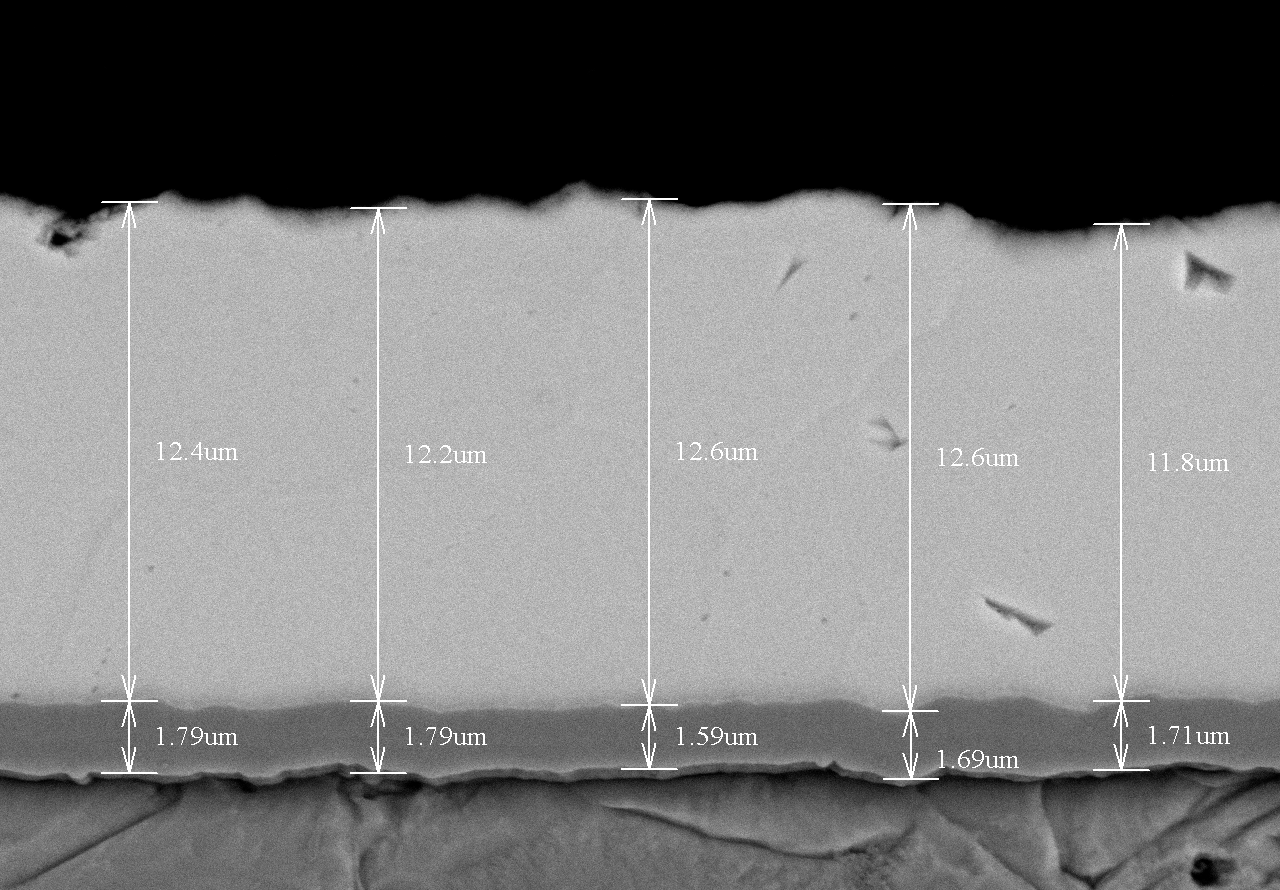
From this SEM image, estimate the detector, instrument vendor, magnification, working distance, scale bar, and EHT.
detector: BSE3D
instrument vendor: Hitachi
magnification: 4 K X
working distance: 8.7 mm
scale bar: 10000 nm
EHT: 15 kV